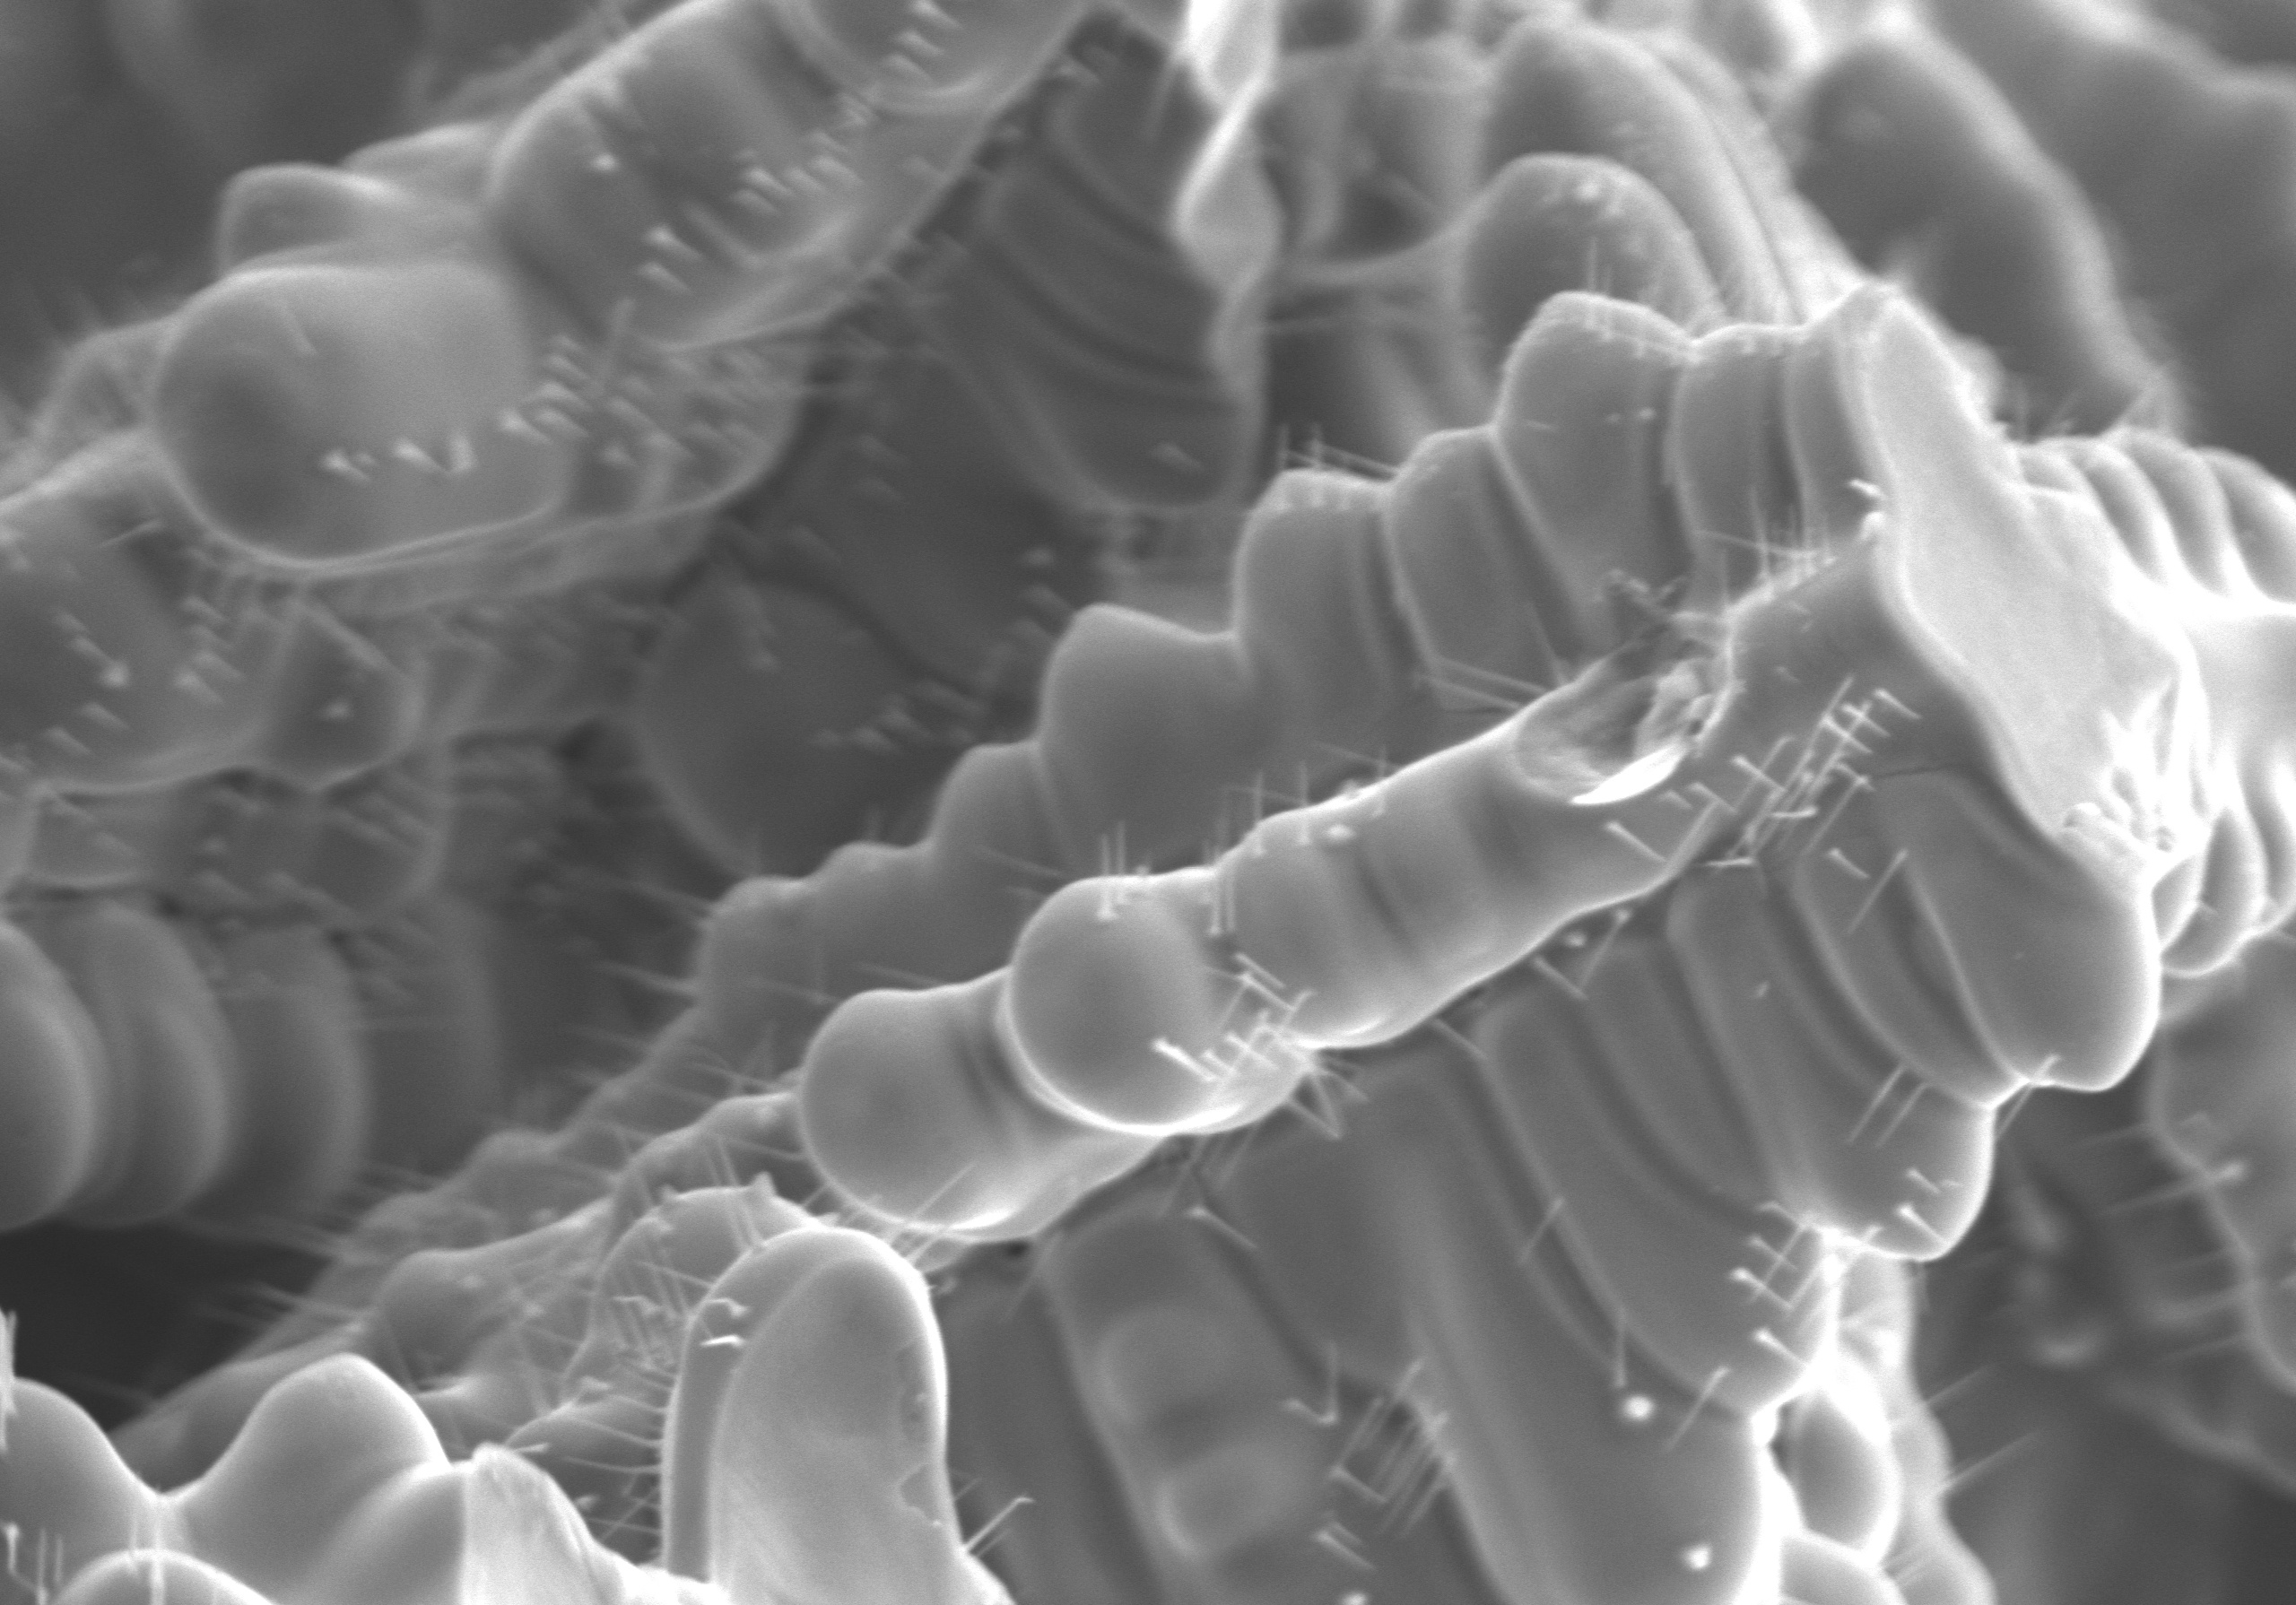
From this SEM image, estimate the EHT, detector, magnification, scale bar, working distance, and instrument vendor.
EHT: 15 kV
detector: SE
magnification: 1.7 K X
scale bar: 30000 nm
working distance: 10.2 mm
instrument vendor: Hitachi